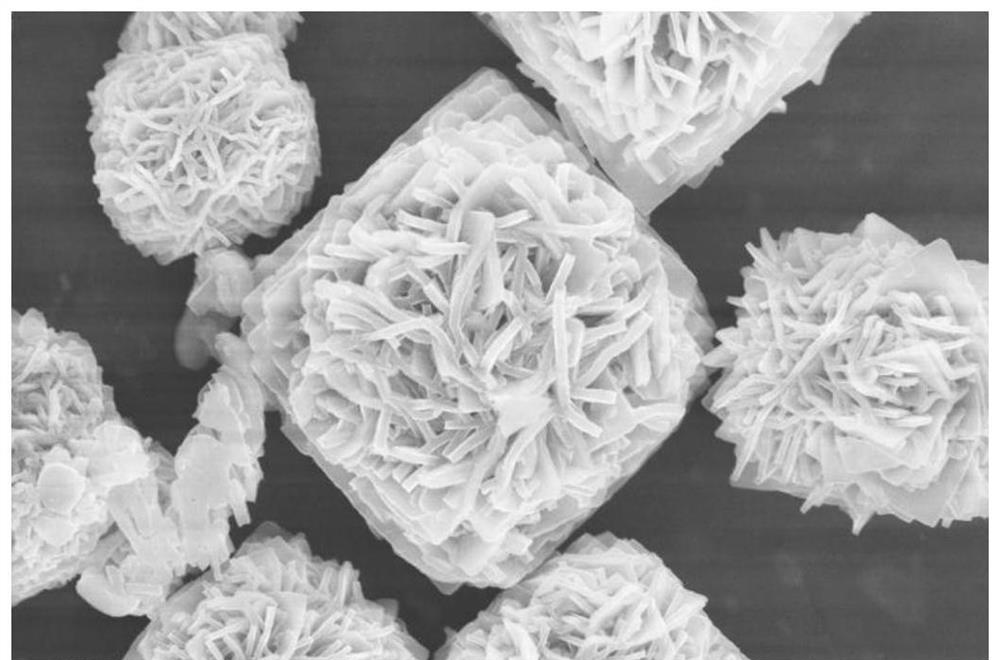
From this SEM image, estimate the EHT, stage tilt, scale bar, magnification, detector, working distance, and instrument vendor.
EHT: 15 kV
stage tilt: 0°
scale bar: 5000 nm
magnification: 15 K X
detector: TLD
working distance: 4.4 mm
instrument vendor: FEI